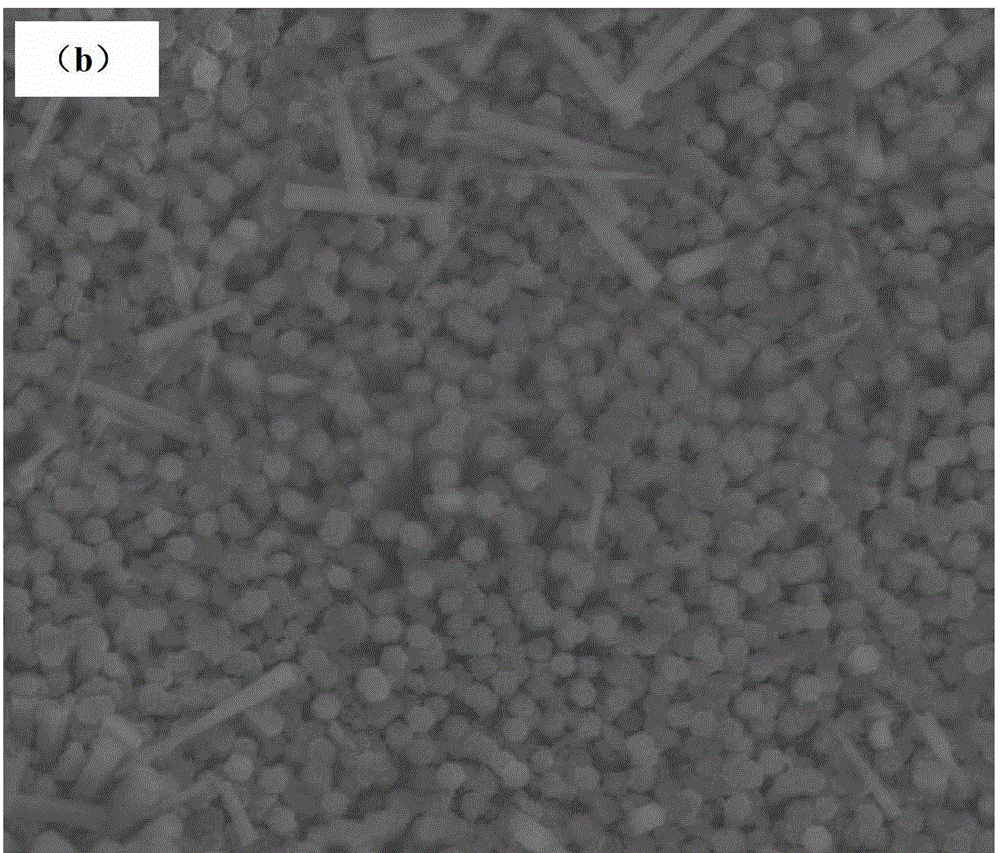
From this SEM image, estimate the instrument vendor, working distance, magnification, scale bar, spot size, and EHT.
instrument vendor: FEI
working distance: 13.8 mm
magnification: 6 K X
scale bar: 10000 nm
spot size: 3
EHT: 15 kV